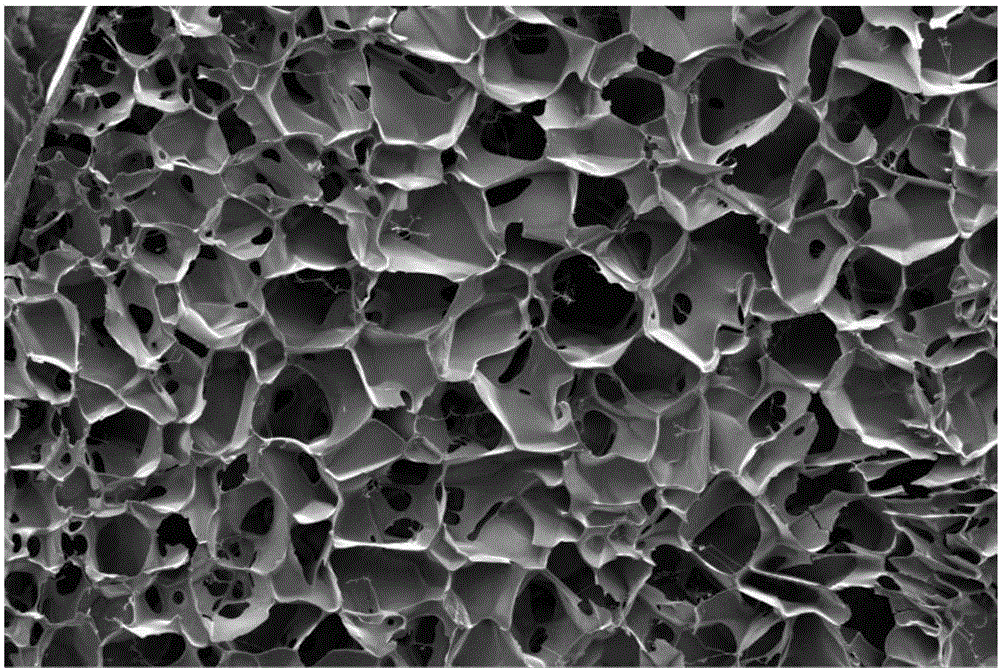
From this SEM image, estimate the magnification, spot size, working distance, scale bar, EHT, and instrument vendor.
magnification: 0.2 K X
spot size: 4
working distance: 14.4 mm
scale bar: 1000000 nm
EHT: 10 kV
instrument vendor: FEI